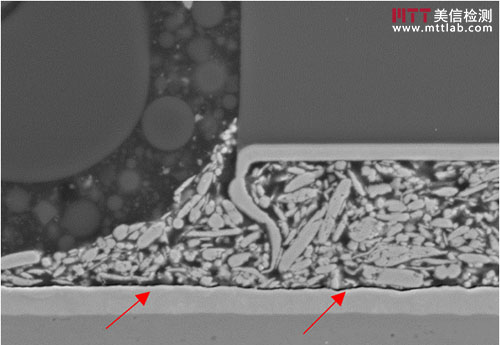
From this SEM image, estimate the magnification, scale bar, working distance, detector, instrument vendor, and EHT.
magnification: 3 K X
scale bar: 10000 nm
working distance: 10 mm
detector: SE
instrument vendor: Hitachi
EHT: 15 kV